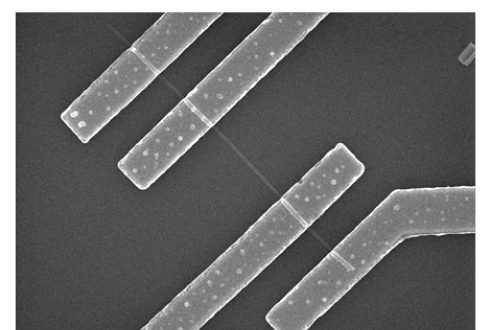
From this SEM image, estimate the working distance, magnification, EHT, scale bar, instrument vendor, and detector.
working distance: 5 mm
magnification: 10 K X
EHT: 10 kV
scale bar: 5000 nm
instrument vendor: Hitachi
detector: SE(M)